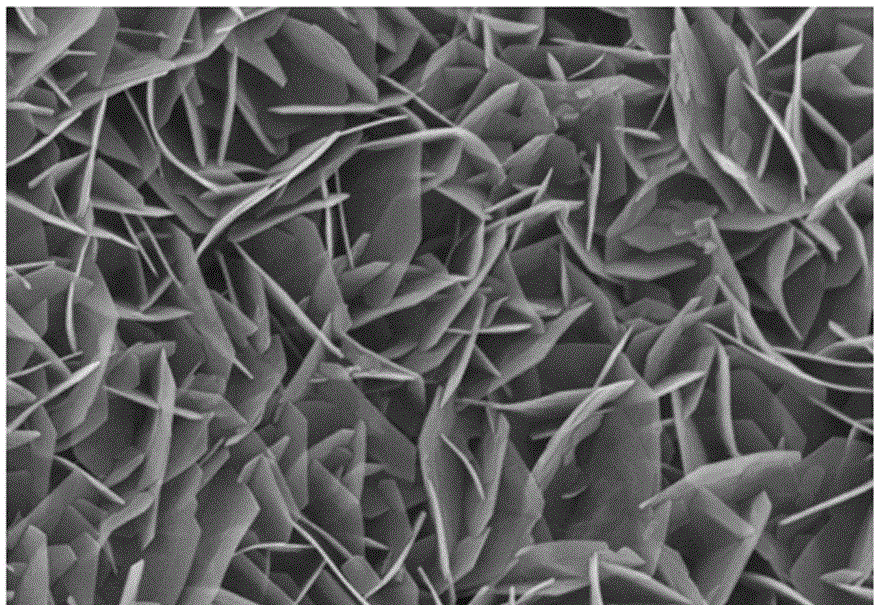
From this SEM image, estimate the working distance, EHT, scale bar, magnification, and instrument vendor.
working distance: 11.7 mm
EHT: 15 kV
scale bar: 3000 nm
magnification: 18 K X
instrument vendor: Hitachi